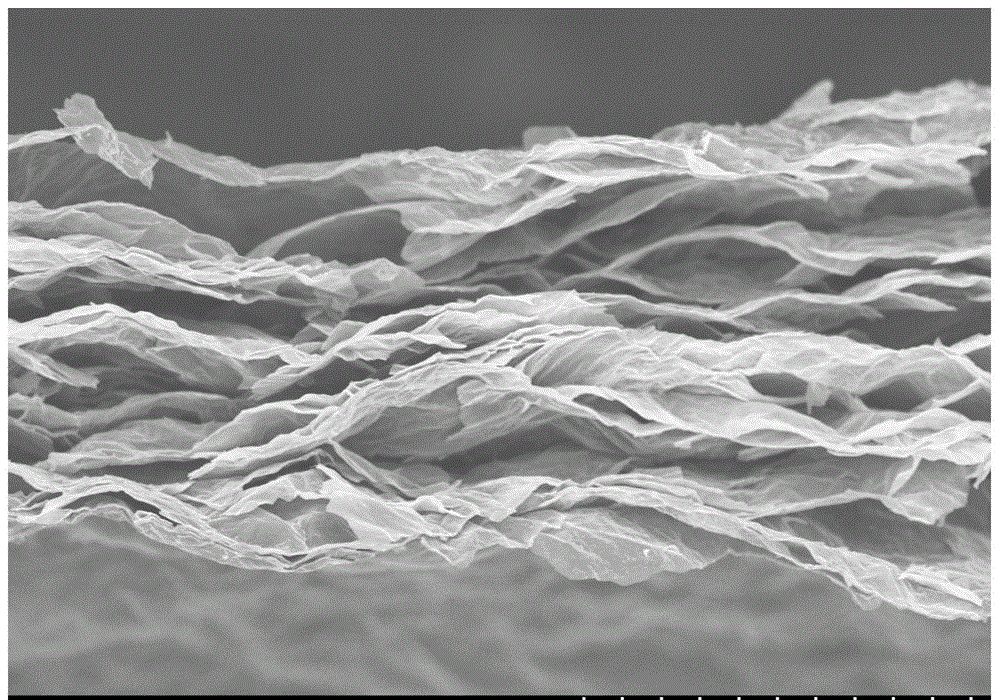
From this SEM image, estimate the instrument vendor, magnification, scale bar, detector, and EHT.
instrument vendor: Hitachi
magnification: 1 K X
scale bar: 50000 nm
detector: SE(U)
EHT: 8 kV